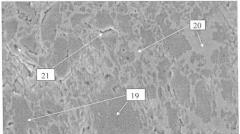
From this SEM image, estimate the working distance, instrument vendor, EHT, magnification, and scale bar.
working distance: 25 mm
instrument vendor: Zeiss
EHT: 20 kV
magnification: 1 K X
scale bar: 1000 nm